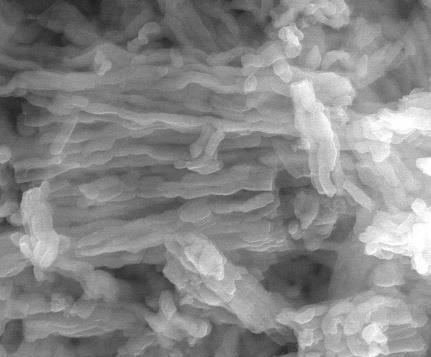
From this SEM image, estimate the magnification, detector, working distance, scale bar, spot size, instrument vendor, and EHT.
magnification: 16 K X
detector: LFD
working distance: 10 mm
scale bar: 5000 nm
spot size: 4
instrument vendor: FEI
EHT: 30 kV